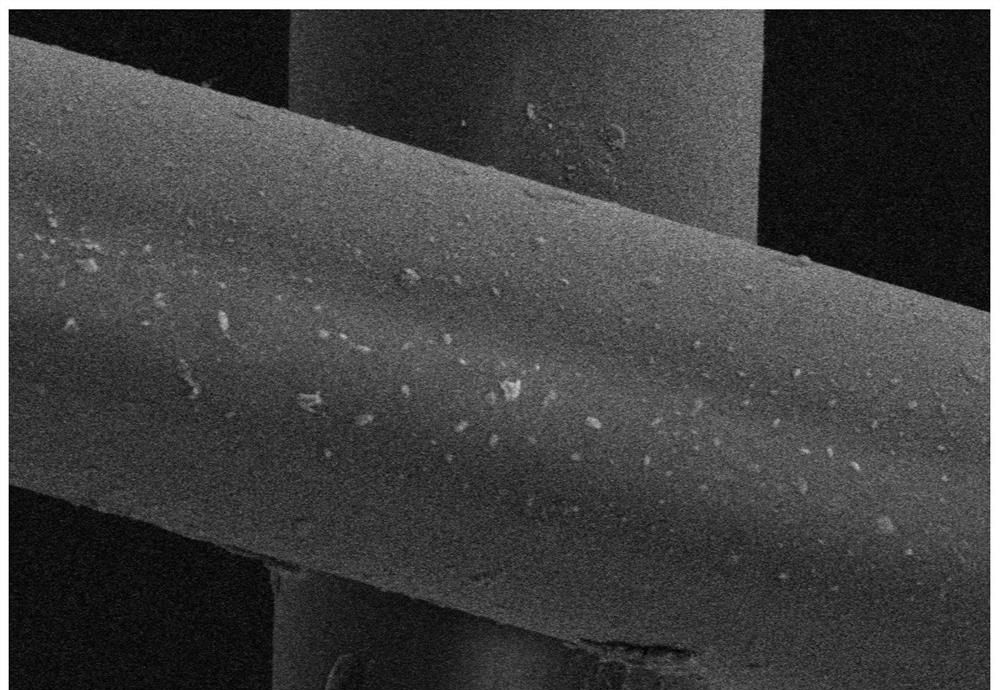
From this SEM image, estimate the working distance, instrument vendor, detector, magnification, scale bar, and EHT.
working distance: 9.8 mm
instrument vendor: Hitachi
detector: SE(UL)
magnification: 5 K X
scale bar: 10000 nm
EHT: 3 kV